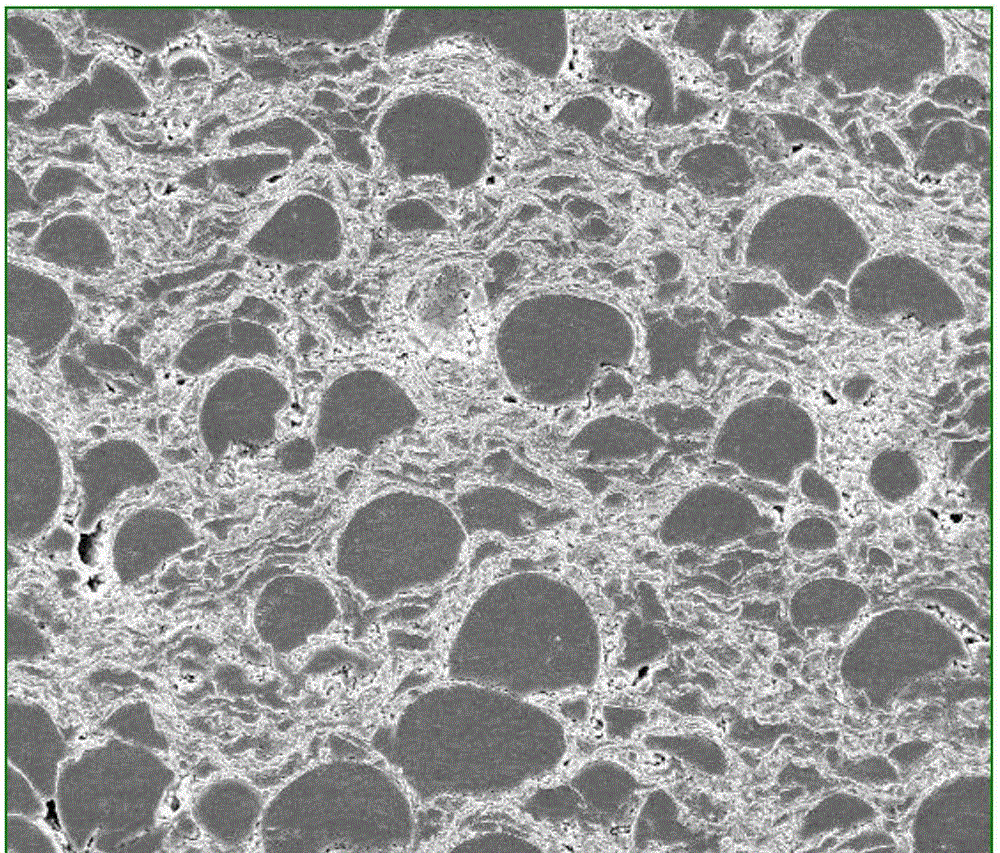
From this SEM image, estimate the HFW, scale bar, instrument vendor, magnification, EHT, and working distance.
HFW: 298 µm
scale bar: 100000 nm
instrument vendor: FEI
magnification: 1 K X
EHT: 20 kV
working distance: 9.2 mm